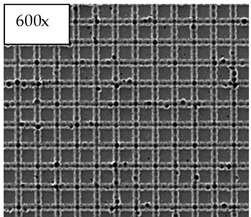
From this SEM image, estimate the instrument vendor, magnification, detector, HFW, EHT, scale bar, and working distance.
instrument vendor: FEI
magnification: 0.6 K X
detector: ETD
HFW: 497 µm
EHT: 30 kV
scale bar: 100000 nm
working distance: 6.8 mm